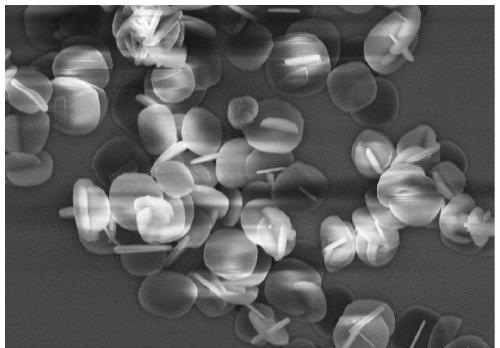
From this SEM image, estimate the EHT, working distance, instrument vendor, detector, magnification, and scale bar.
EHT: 5 kV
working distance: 9.7 mm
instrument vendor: Hitachi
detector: SE(U)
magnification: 5 K X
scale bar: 10000 nm